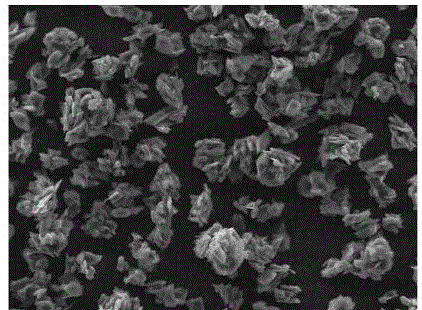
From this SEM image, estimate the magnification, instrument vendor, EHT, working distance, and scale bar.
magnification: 1 K X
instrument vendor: Tescan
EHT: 20 kV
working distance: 14.87 mm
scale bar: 50000 nm